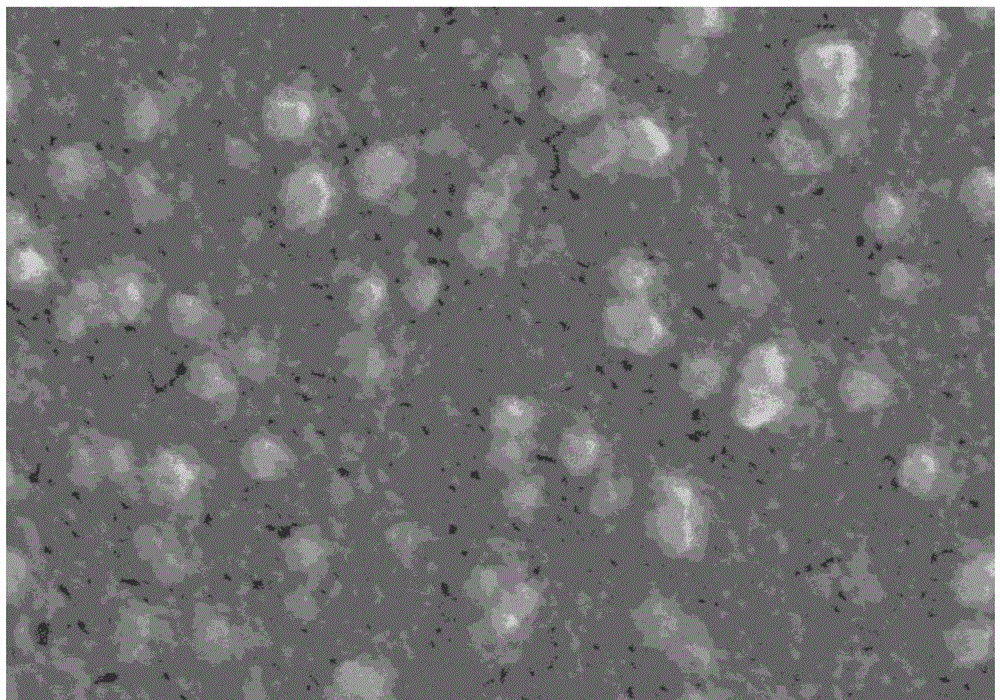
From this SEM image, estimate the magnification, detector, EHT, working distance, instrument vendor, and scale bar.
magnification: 50 K X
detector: SE(M)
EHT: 15 kV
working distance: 27.1 mm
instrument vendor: Hitachi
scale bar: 1000 nm